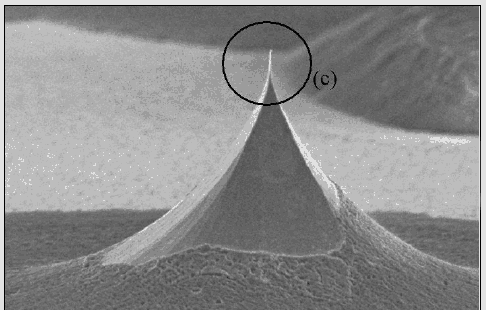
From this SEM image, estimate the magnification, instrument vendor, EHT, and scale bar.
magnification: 2 K X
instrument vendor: JEOL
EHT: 5 kV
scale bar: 10000 nm